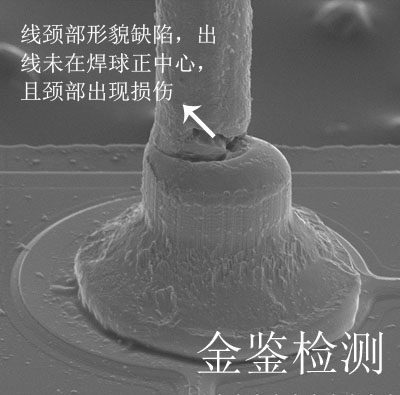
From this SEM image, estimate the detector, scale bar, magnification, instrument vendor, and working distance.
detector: SE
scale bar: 50000 nm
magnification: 0.8 K X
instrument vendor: Hitachi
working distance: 20.6 mm